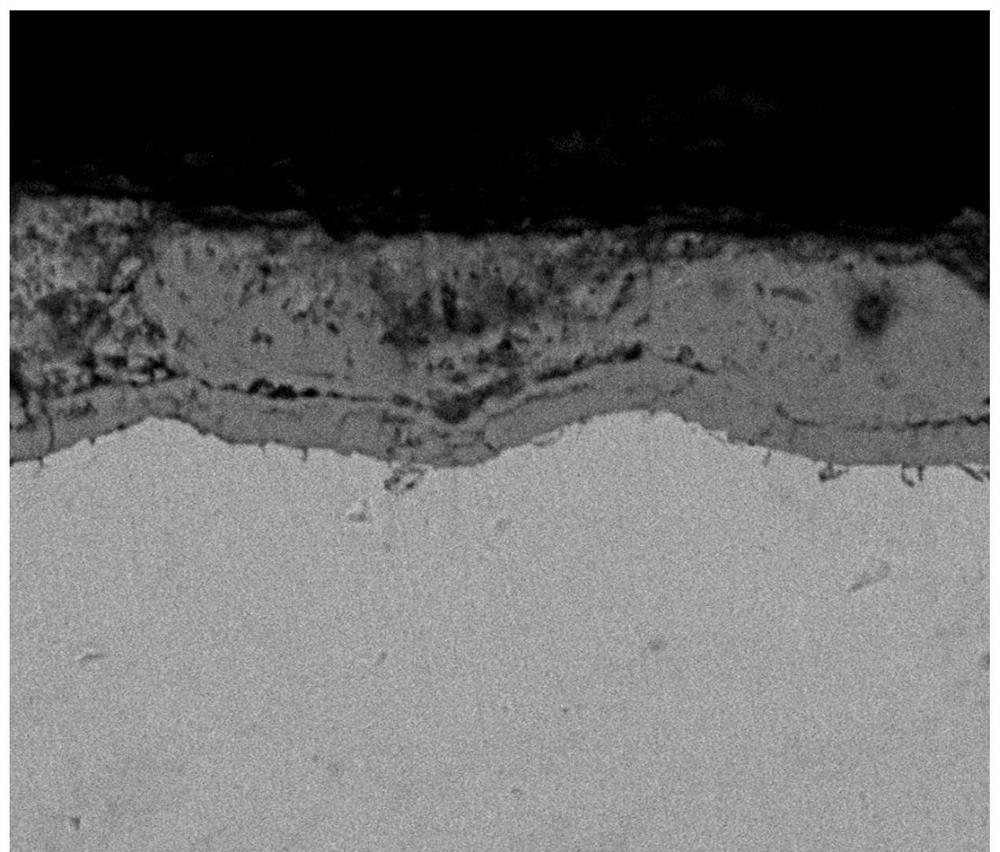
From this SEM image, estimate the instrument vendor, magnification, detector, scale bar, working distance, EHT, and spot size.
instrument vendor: FEI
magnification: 2 K X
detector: SSD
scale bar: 20000 nm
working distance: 11.5 mm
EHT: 15 kV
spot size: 6